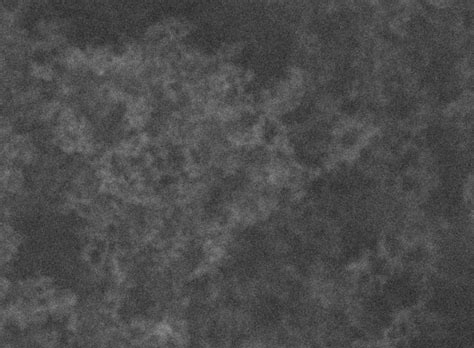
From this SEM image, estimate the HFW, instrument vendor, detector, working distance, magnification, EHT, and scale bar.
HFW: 2.77 µm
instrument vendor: Tescan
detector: SE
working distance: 8.02 mm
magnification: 150 K X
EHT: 30 kV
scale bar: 500 nm